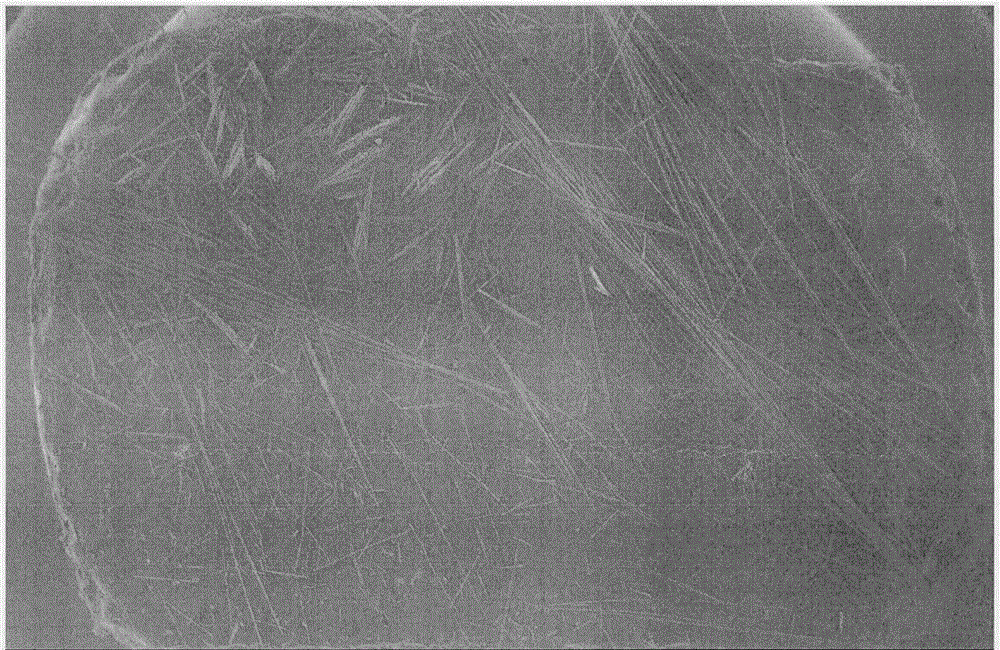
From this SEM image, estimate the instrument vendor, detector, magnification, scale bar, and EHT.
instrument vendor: JEOL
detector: SEI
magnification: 0.02 K X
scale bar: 1000000 nm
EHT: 20 kV